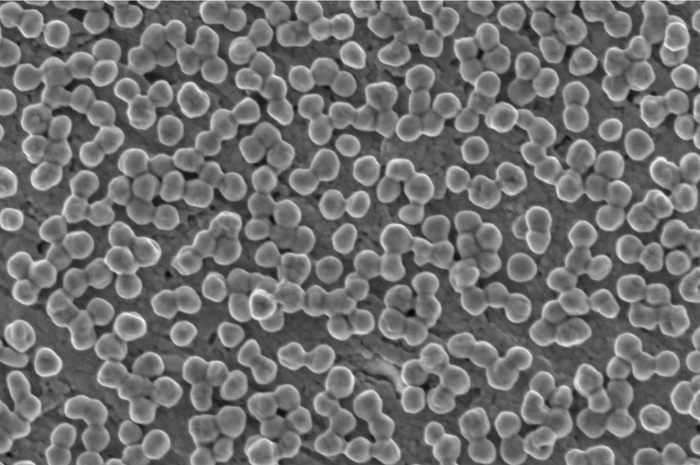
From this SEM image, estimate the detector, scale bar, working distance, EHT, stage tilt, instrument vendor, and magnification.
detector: TLD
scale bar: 1000 nm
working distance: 4 mm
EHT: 2 kV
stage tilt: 0°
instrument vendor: FEI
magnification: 80 K X